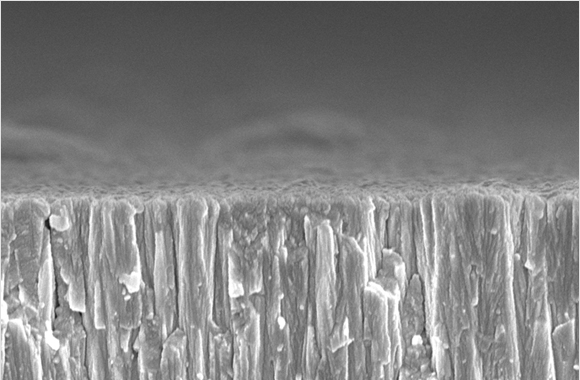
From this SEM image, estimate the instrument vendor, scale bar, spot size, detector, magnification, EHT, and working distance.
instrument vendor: FEI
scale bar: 1000 nm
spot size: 3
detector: TLD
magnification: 50 K X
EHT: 5 kV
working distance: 5.9 mm